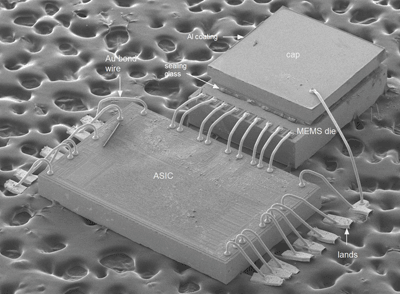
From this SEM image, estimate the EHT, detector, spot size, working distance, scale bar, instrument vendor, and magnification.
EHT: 10 kV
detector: SE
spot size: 3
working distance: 14.5 mm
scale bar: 500000 nm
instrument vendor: FEI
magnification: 0.035 K X